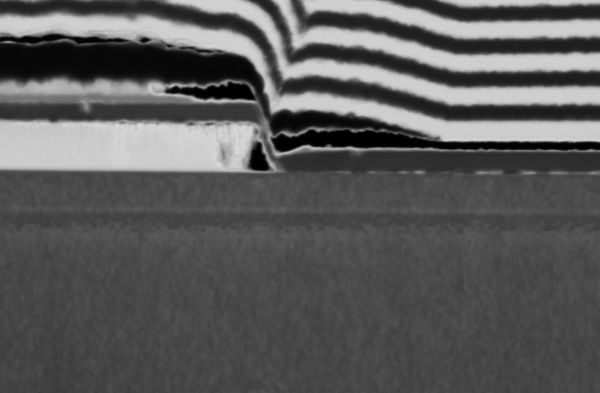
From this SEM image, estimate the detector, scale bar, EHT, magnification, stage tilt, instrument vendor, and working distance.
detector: STEM 3+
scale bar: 300 nm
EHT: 30 kV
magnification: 120 K X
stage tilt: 38°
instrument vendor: FEI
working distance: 7 mm